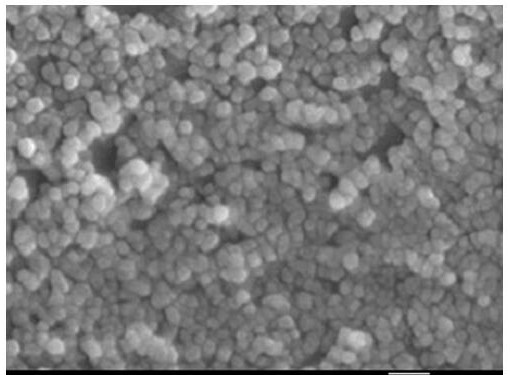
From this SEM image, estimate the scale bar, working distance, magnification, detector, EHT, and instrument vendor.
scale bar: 100 nm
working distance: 16 mm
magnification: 100 K X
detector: SEI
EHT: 15 kV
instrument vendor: JEOL